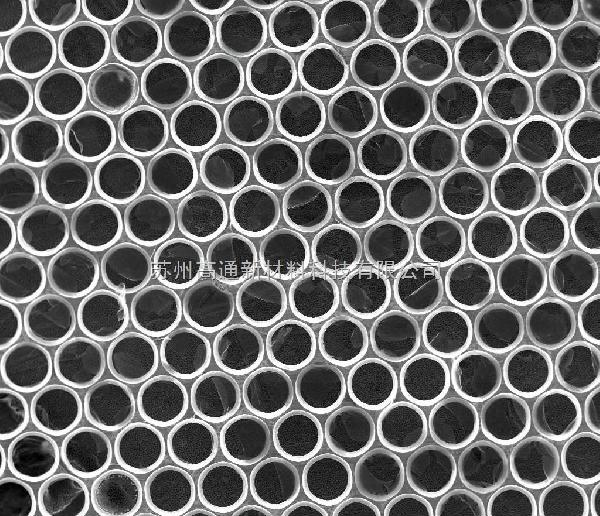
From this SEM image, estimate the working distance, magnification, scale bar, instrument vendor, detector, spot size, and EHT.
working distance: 12.9 mm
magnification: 0.1 K X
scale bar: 500000 nm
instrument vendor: FEI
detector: ETD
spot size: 3.5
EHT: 20 kV